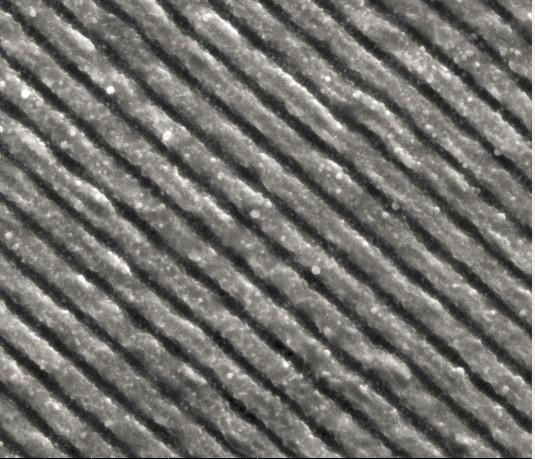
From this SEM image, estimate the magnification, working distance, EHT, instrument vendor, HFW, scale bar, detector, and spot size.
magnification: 10 K X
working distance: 9.9 mm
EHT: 20 kV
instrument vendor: FEI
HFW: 13.52 µm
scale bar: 5000 nm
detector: ETD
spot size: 3